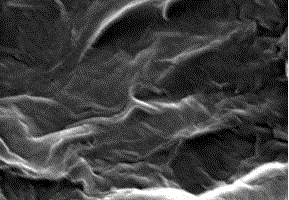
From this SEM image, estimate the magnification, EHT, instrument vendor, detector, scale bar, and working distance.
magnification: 100 K X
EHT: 15 kV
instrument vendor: Hitachi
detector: SE(UL)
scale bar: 500 nm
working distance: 15.5 mm